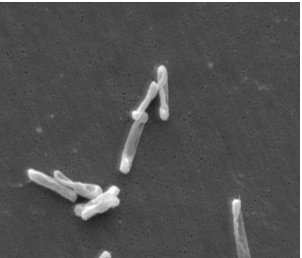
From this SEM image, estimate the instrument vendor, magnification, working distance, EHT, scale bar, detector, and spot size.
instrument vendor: FEI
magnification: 4.753 K X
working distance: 33.8 mm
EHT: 10 kV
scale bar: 5000 nm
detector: SE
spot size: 3